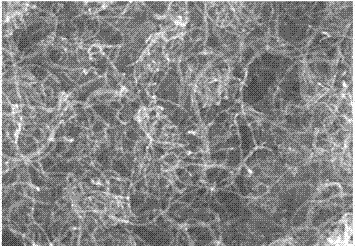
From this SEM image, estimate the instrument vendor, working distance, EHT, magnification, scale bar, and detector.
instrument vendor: Hitachi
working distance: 7.1 mm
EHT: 15 kV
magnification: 60 K X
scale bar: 500 nm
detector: SE(U)